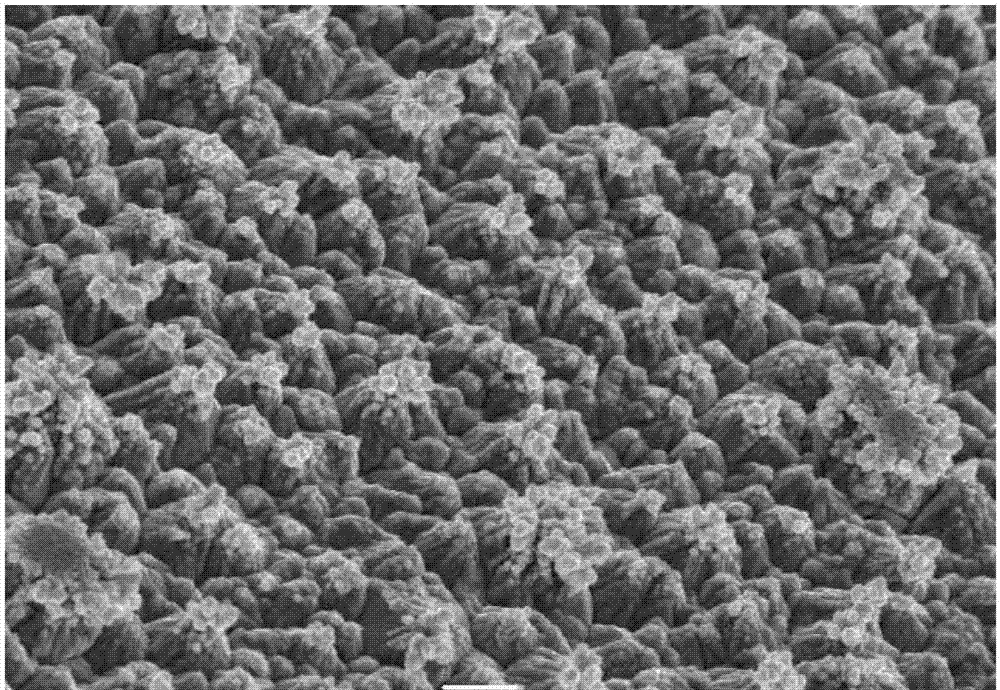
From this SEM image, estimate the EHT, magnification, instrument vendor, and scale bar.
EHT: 30 kV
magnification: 1 K X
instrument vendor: JEOL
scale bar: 10000 nm